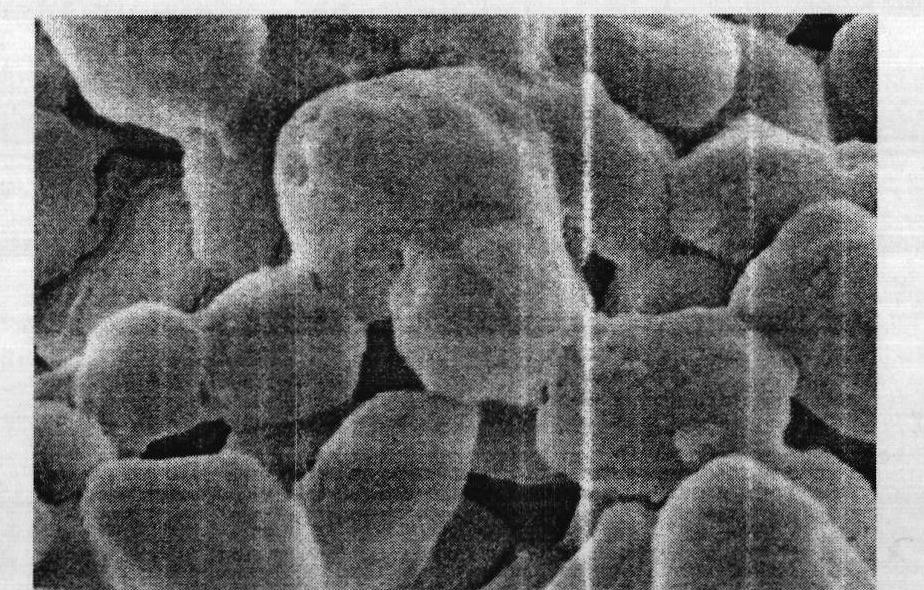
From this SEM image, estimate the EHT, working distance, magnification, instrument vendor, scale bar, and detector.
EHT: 3 kV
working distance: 7.2 mm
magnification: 80 K X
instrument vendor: Hitachi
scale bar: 500 nm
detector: SE(M)